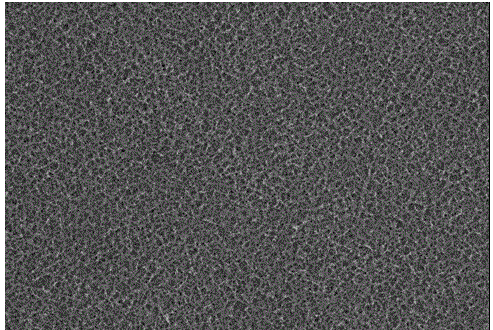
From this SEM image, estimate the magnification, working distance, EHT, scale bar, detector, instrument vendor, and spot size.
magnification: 1 K X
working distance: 10 mm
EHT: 10 kV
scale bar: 10000 nm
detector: SEI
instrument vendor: JEOL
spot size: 30